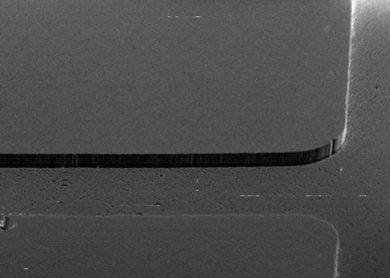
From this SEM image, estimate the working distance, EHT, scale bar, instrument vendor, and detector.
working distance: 2.6 mm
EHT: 15 kV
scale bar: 10000 nm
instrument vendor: Zeiss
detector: SE2